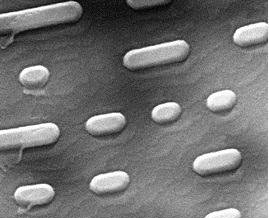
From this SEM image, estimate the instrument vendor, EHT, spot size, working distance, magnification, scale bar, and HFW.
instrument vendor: FEI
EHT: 10 kV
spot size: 3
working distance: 10.4 mm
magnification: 20 K X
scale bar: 2000 nm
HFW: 7.46 µm